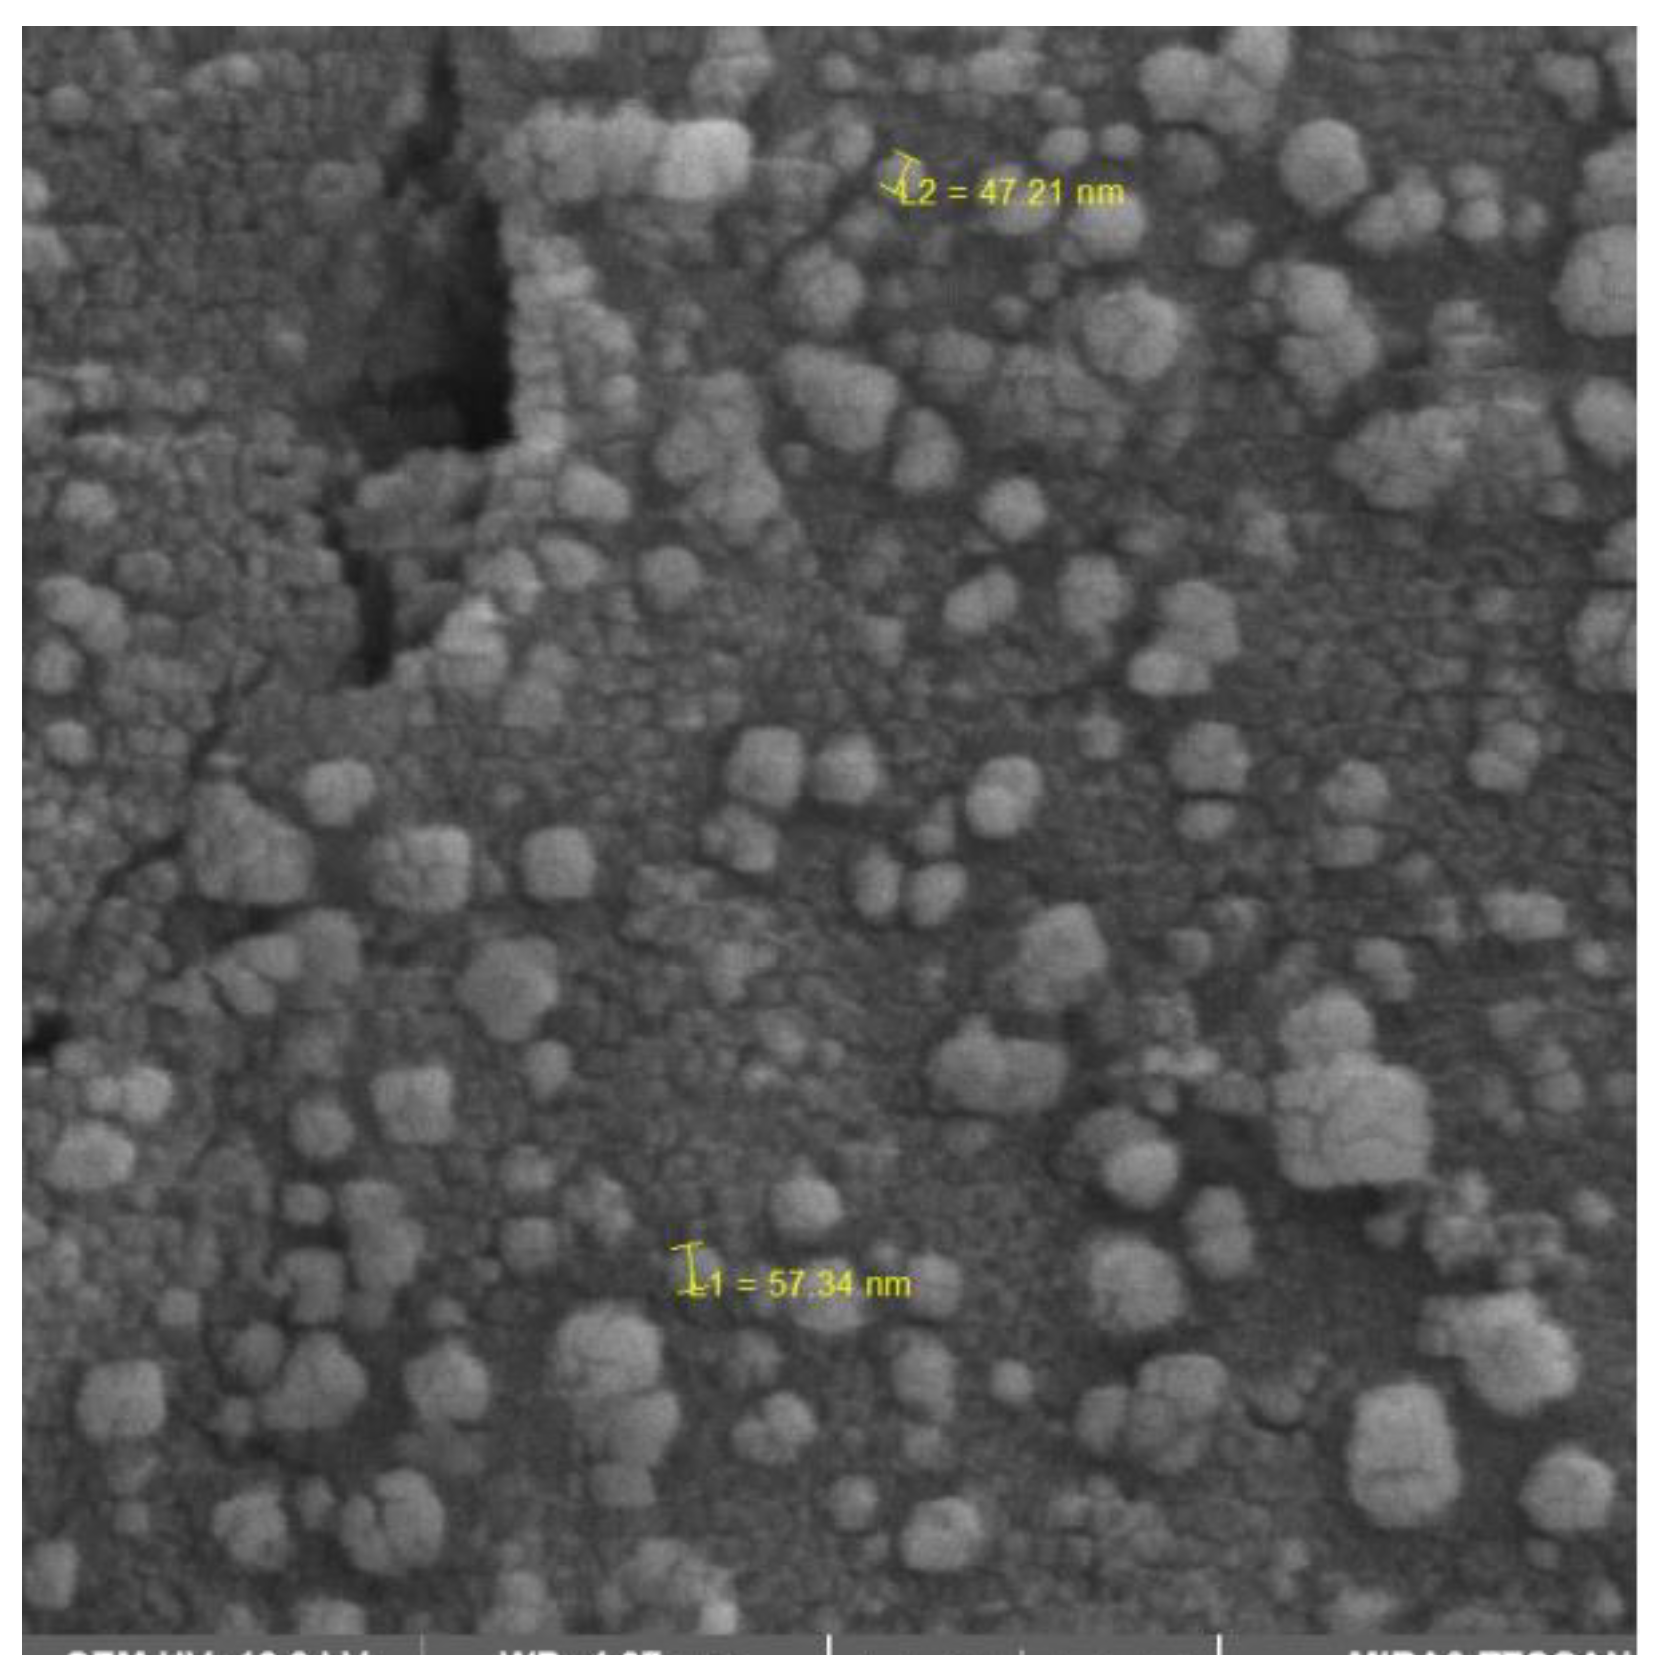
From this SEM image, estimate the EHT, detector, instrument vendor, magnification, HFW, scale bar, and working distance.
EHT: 10 kV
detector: SE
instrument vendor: Tescan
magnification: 100 K X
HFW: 2.08 µm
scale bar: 500 nm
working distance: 4.97 mm